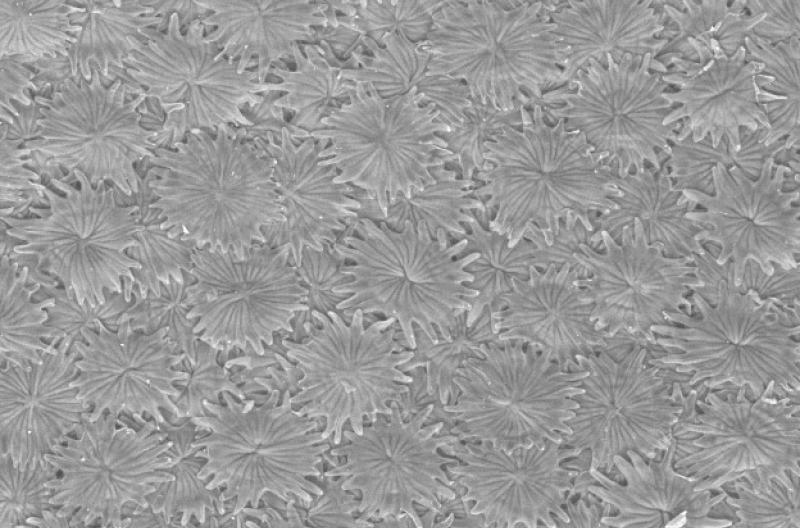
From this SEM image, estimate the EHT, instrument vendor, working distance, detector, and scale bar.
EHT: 20.16 kV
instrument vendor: Zeiss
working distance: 12.5 mm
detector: NTS BSD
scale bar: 100000 nm